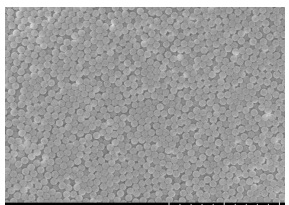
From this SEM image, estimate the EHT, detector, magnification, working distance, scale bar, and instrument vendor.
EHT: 15 kV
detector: SE(UL)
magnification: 10 K X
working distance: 10.7 mm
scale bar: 5000 nm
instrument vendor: Hitachi